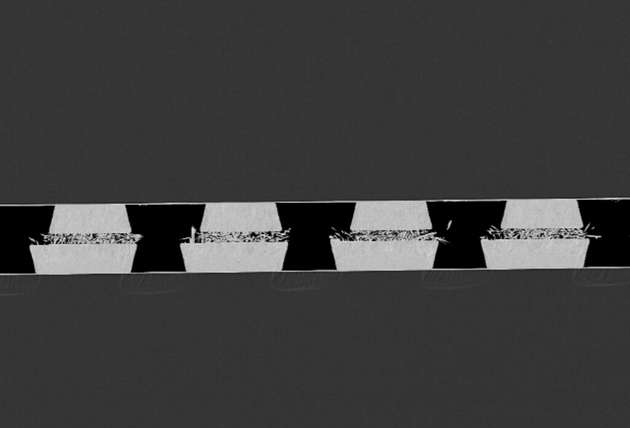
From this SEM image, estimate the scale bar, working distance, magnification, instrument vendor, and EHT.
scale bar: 20000 nm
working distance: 8 mm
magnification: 0.5 K X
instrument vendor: Zeiss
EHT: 10 kV